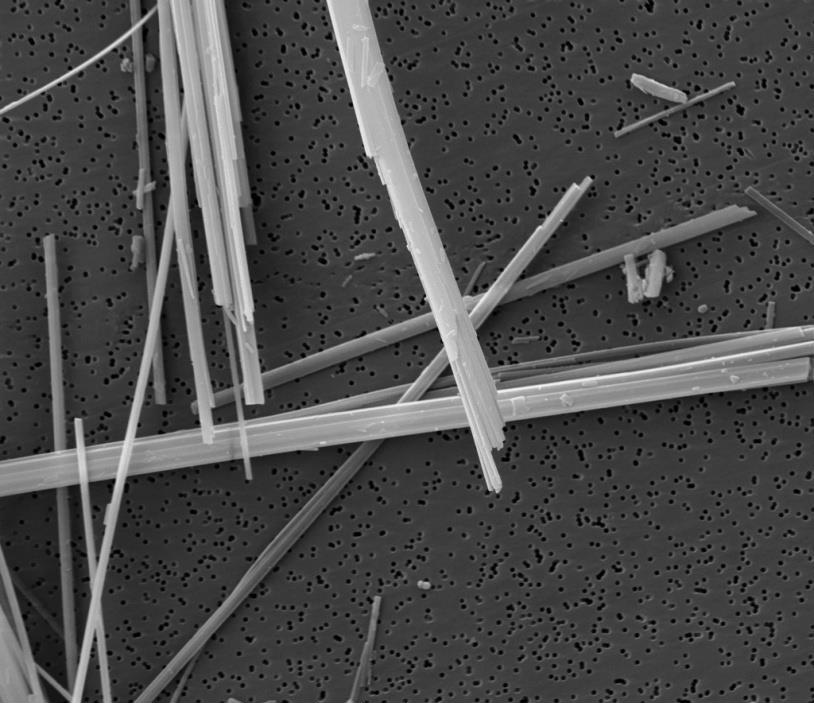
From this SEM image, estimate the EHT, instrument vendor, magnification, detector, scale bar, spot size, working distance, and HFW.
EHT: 20 kV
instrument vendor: FEI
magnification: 10 K X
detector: ETD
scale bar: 10000 nm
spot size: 3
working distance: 10.9 mm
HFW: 27.04 µm